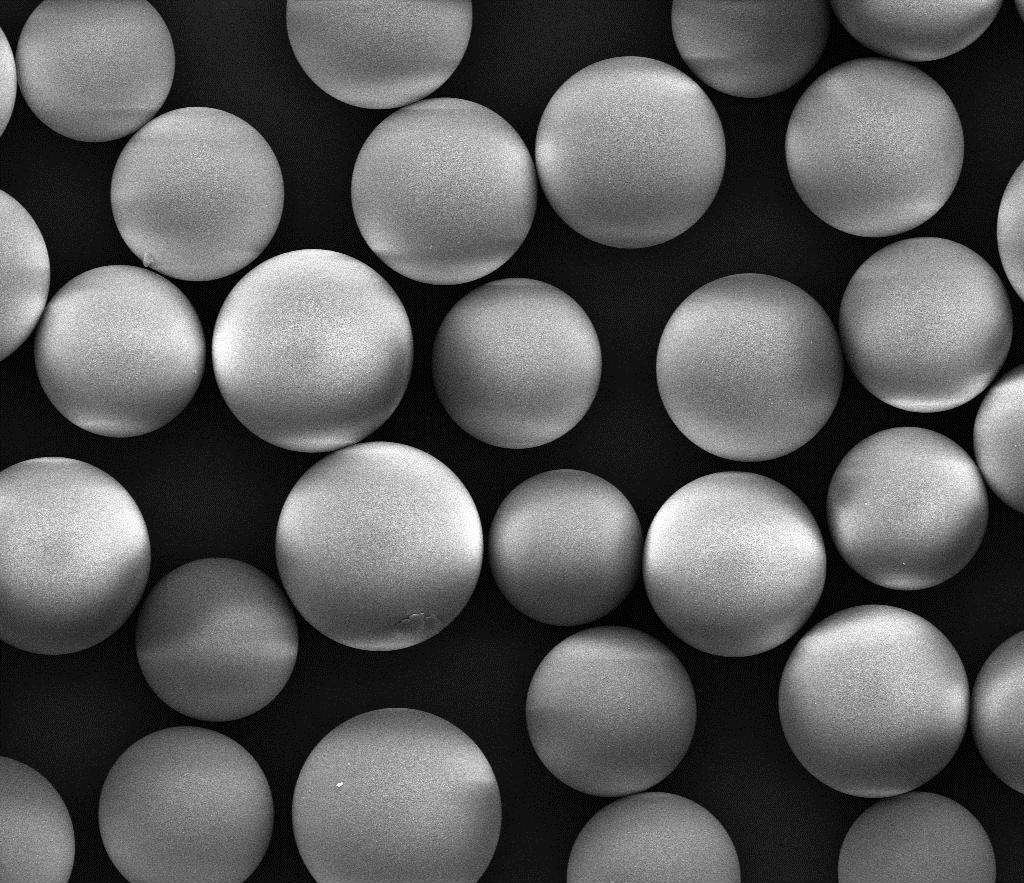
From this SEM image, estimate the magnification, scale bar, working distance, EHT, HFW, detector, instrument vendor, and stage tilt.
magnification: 2 K X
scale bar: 50000 nm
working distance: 8.5 mm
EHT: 20 kV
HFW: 149 µm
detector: ETD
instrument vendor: FEI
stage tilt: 0°